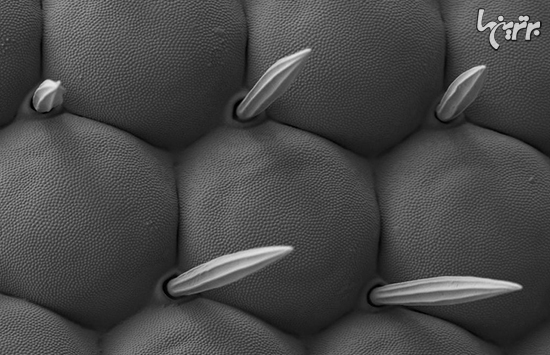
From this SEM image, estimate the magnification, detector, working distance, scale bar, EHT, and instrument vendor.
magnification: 3 K X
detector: SE2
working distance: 4.2 mm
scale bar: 2000 nm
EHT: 1 kV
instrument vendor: Zeiss